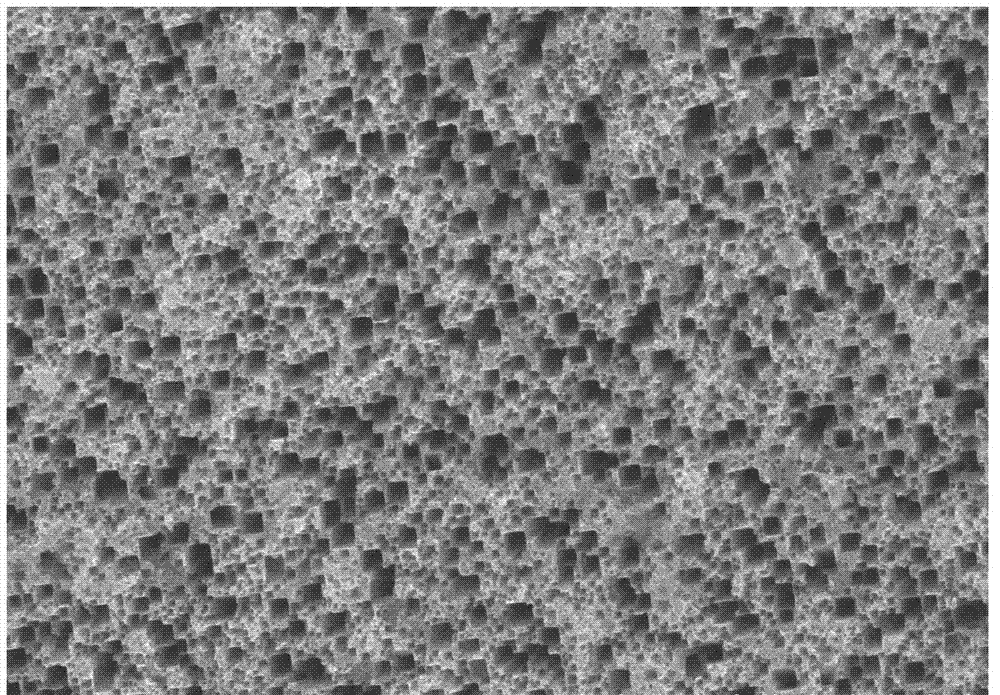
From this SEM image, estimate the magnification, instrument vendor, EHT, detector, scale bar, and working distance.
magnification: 5 K X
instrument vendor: Hitachi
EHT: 20 kV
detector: SE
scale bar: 10000 nm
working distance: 9.4 mm